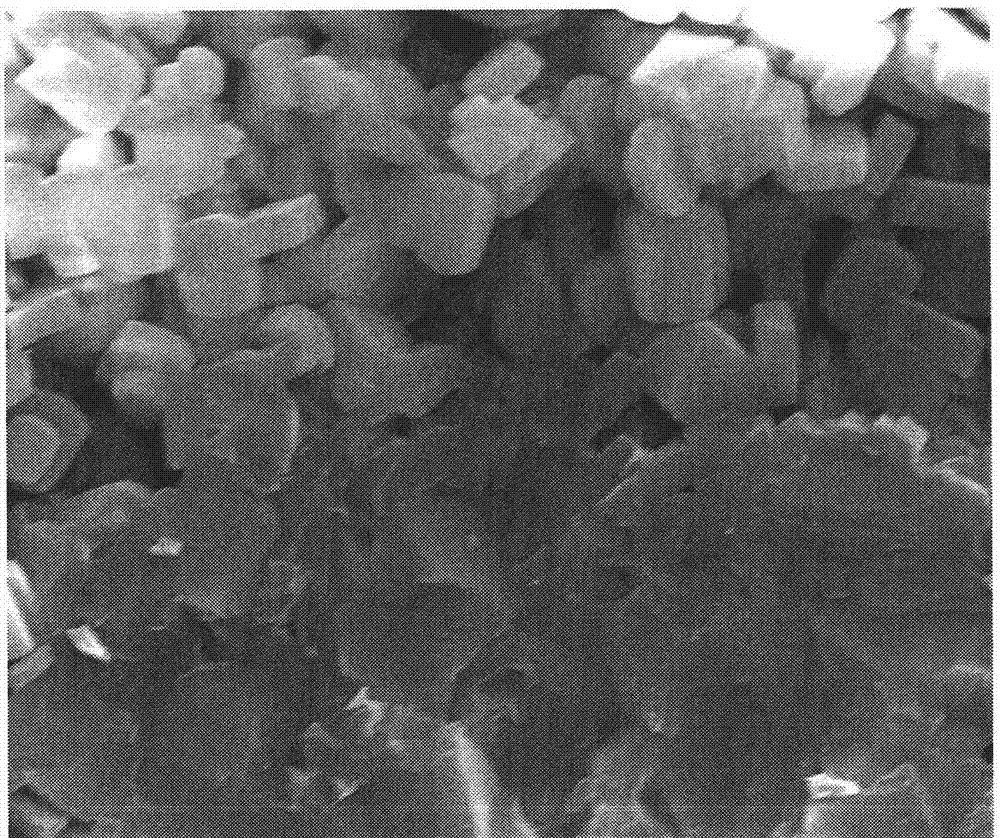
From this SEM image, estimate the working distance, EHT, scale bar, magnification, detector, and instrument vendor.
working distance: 15.9 mm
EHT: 20 kV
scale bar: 10000 nm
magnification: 10 K X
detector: ETD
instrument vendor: FEI